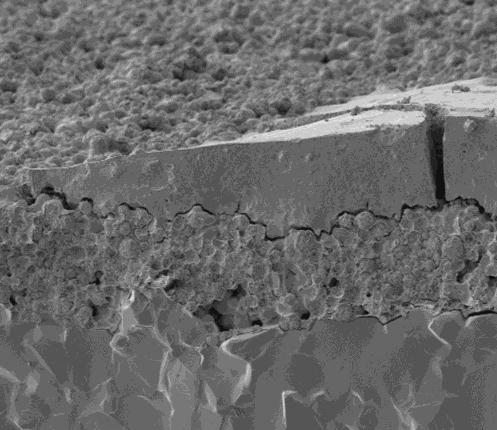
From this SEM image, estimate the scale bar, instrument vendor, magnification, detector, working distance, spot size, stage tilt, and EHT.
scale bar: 10000 nm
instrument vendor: FEI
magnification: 6 K X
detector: ETD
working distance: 5.2 mm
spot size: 4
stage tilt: -6°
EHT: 2 kV